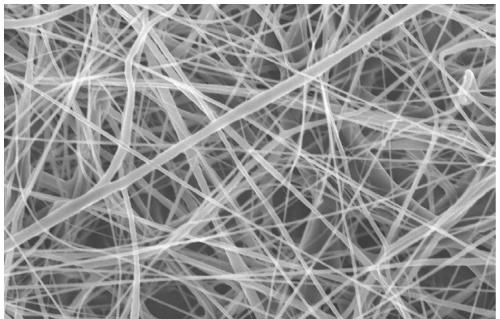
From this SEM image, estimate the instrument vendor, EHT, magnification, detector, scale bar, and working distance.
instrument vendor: Hitachi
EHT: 10 kV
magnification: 2 K X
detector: SE(U)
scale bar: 20000 nm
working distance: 14.2 mm